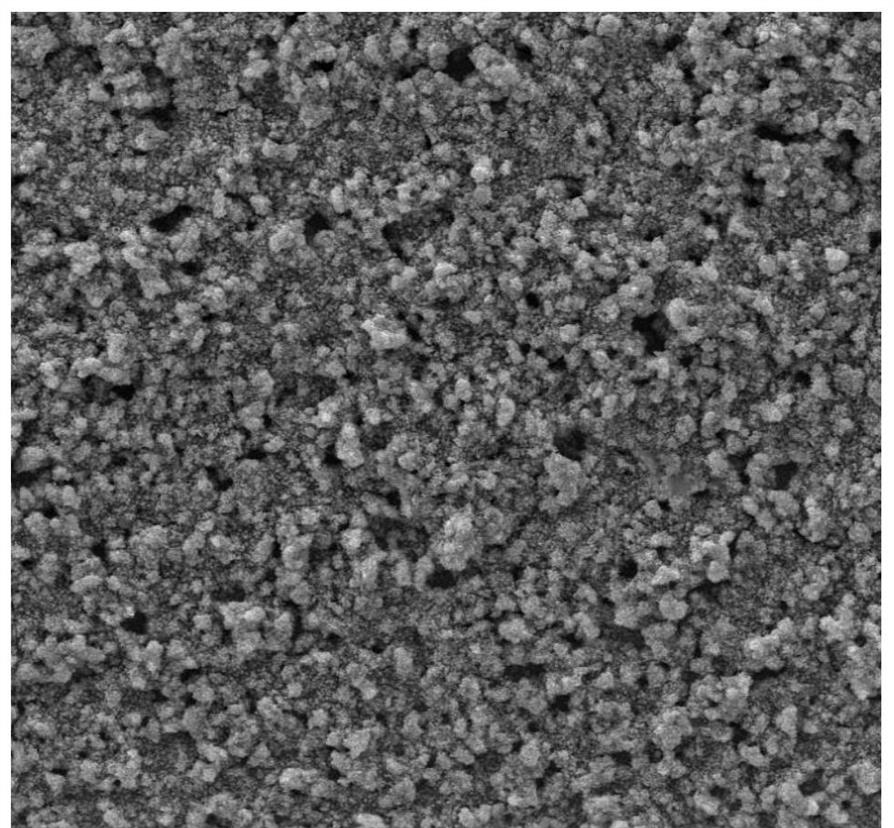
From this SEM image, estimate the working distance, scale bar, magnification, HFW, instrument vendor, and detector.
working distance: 14.61 mm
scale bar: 100000 nm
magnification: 0.5 K X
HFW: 415 µm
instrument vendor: Tescan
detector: SE